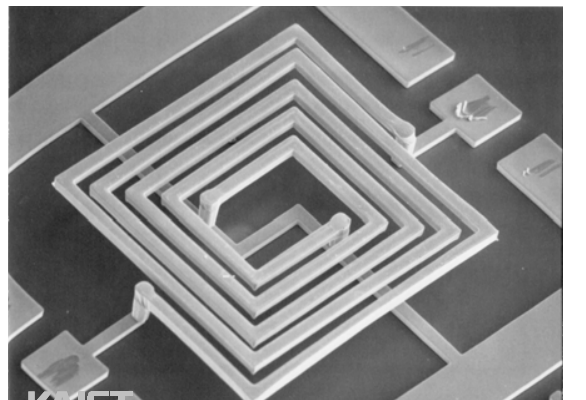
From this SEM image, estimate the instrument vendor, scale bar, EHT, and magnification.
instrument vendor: JEOL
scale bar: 100000 nm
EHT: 20 kV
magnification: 0.1 K X